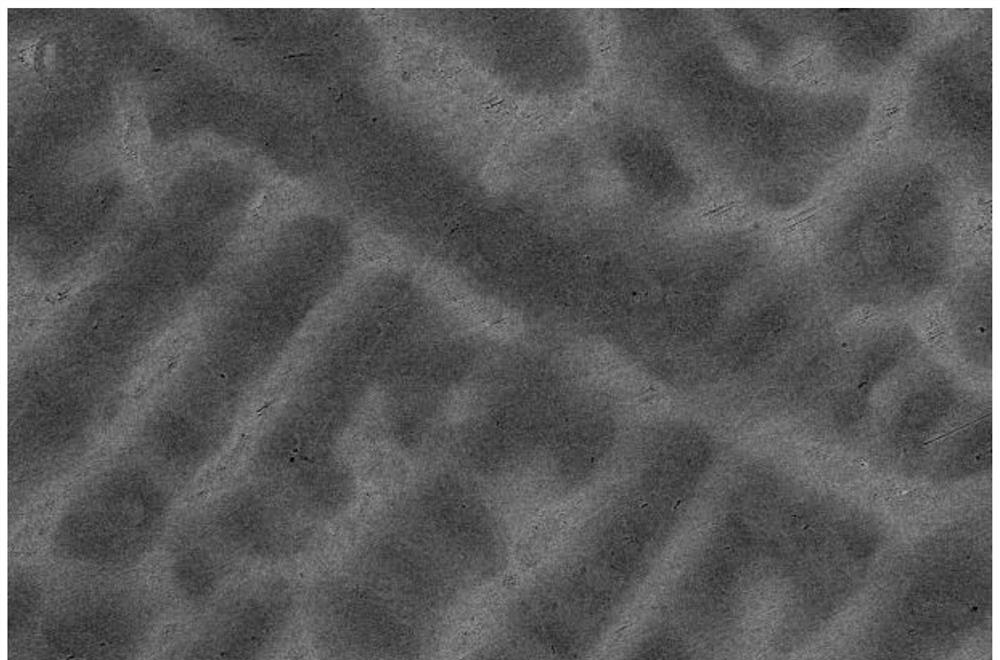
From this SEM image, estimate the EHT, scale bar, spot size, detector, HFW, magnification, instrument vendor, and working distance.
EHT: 25 kV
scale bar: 100000 nm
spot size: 5.5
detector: CBS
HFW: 414 µm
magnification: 0.5 K X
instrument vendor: FEI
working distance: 15.9 mm